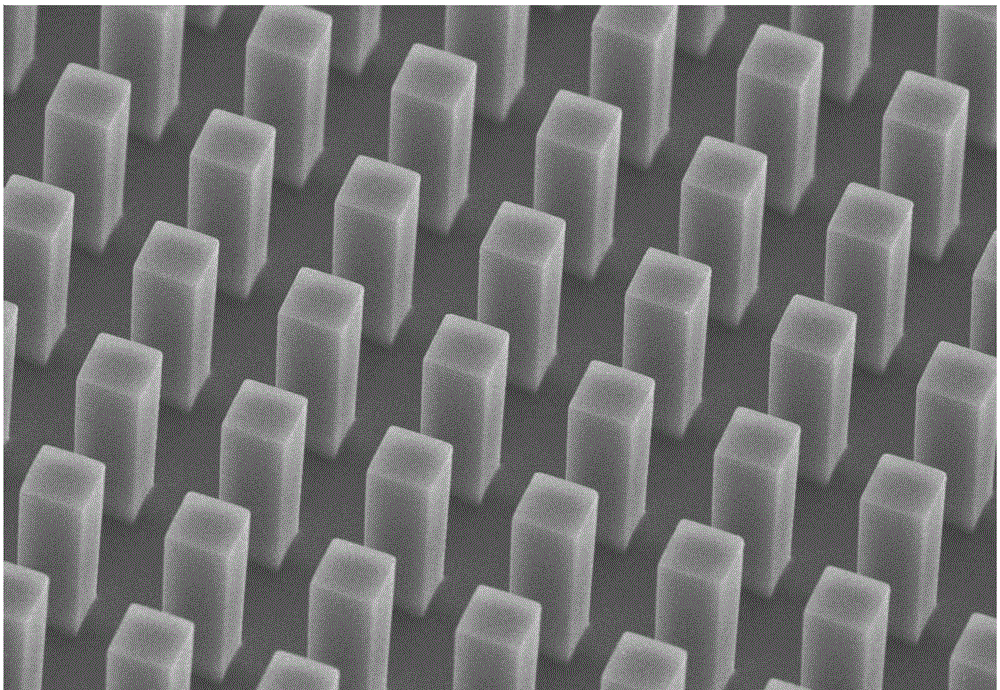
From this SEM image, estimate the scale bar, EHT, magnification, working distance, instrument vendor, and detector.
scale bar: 50000 nm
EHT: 15 kV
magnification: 1 K X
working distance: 7.9 mm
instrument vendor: Hitachi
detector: SE(UL)